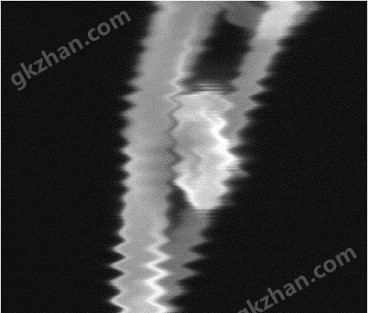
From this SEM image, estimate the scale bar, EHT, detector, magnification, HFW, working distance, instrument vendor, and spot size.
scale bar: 500 nm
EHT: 30 kV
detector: ETD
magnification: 100 K X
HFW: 1.49 µm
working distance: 10 mm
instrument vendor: FEI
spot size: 7